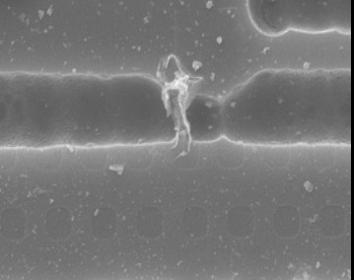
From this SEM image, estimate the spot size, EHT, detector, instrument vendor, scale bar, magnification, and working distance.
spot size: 3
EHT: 15 kV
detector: SE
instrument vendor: FEI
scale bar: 5000 nm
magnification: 5 K X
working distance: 9.9 mm